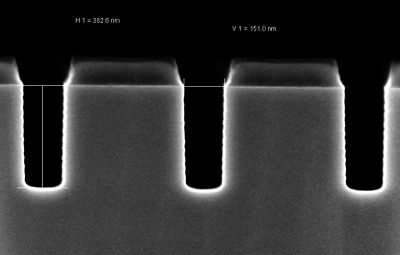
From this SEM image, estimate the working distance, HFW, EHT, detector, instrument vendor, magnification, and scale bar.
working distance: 3.2 mm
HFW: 1.501 µm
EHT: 2 kV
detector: InLens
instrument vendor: Zeiss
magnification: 200 K X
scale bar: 100 nm